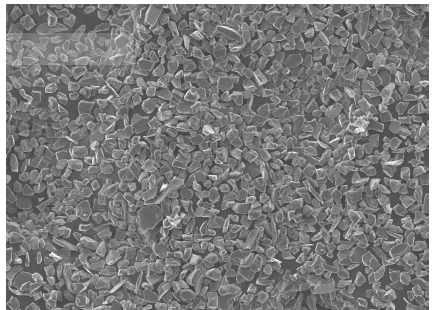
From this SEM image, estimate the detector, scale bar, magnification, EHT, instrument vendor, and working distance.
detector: SEI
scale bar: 100000 nm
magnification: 0.2 K X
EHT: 15 kV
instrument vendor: JEOL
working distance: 19.3 mm